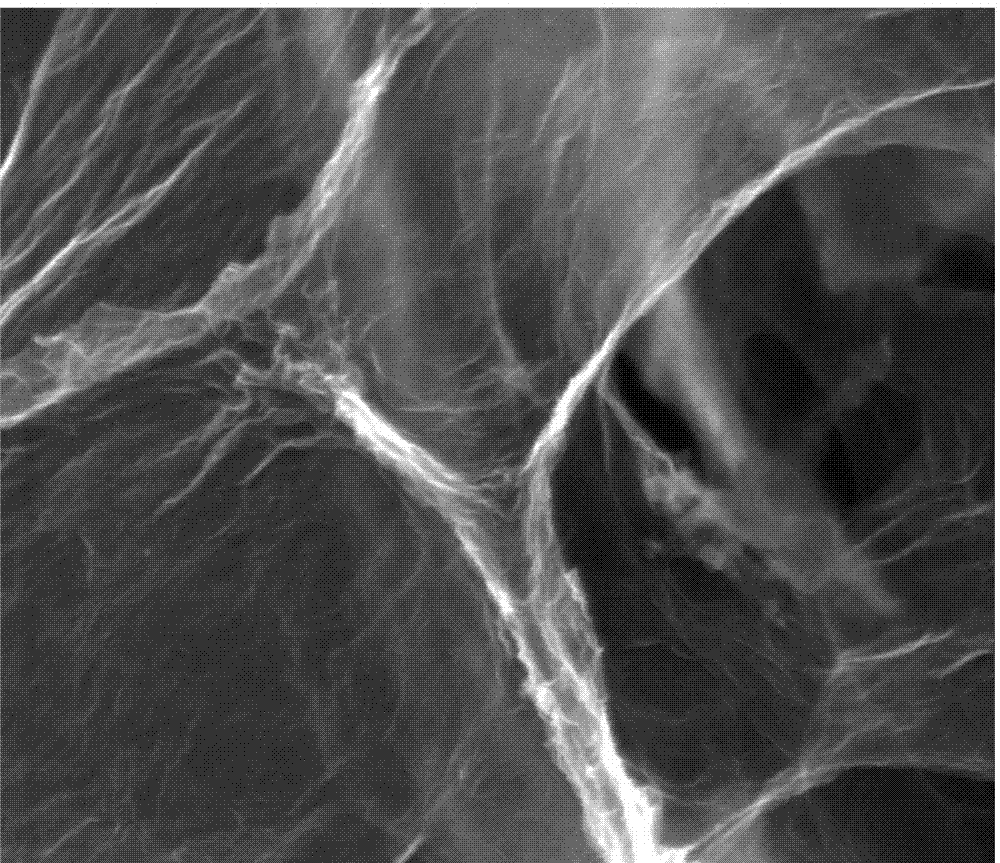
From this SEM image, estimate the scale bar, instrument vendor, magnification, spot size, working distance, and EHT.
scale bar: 1000 nm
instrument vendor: FEI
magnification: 80 K X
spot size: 4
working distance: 9.2 mm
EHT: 30 kV